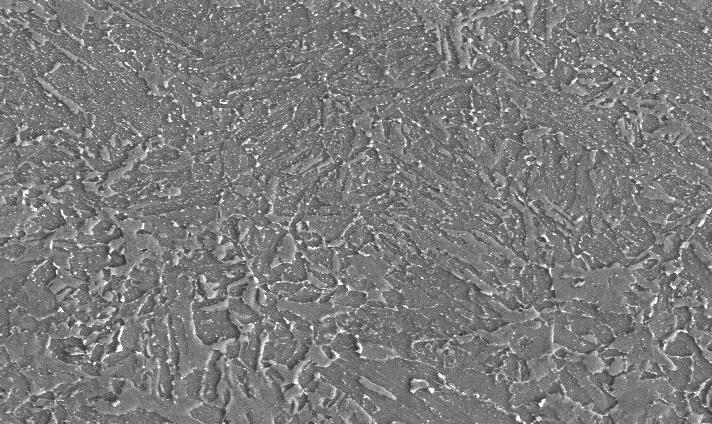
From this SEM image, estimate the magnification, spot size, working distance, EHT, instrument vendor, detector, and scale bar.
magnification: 1 K X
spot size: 4.5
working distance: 8.3 mm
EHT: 20 kV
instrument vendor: FEI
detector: SE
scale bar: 20000 nm